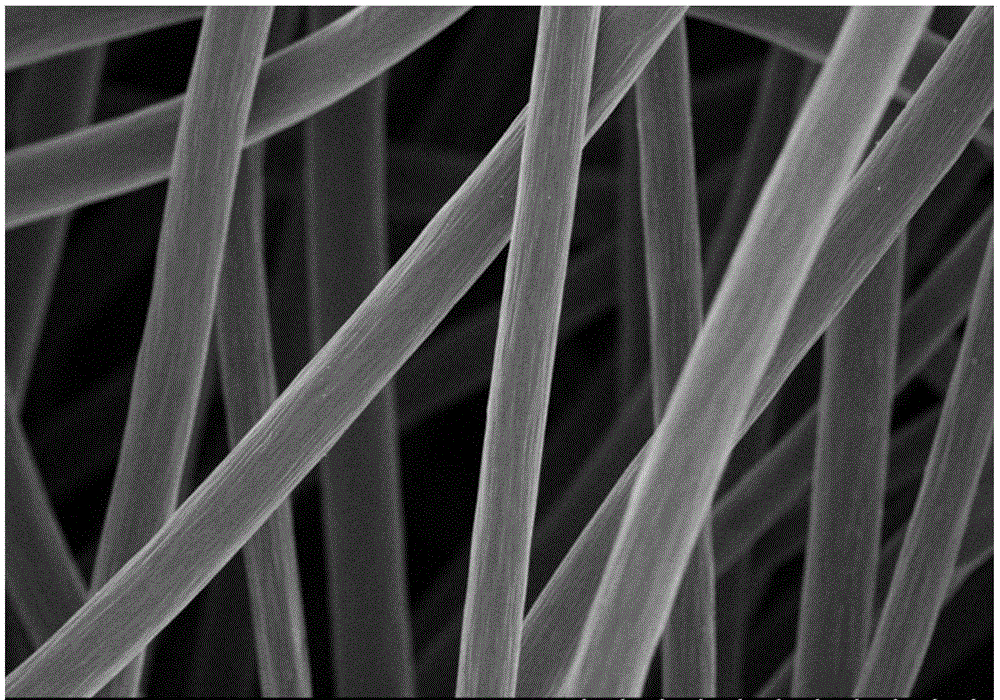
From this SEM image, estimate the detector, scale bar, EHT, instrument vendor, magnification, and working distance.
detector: SE(M)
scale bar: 100000 nm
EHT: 5 kV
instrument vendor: Hitachi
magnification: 0.5 K X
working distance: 8.7 mm